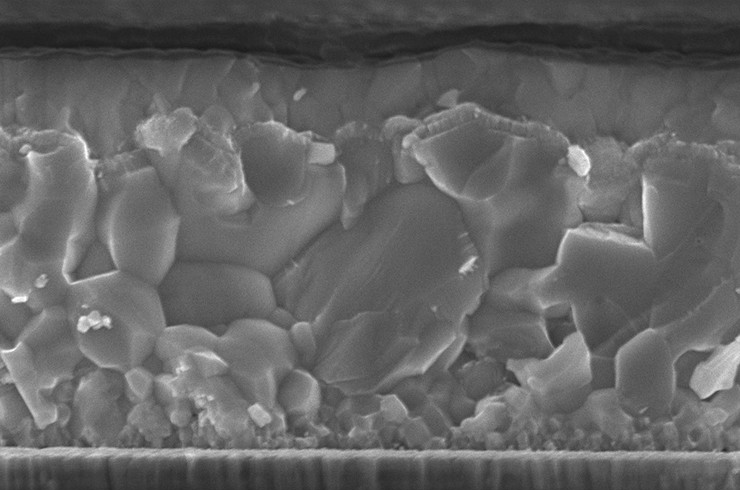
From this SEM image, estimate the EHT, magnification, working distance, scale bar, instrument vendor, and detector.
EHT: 8 kV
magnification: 20.07 K X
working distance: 5.9 mm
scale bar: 400 nm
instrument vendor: Zeiss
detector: InLens